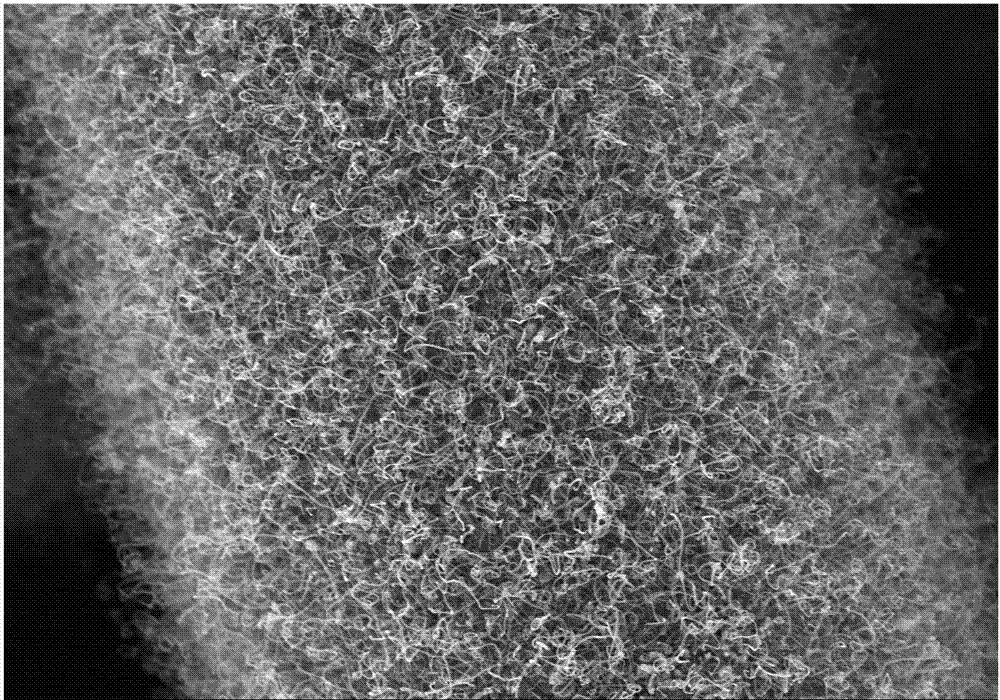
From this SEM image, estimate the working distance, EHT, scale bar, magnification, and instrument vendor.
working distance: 9.2 mm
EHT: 10 kV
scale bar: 20000 nm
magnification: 2 K X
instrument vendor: Hitachi